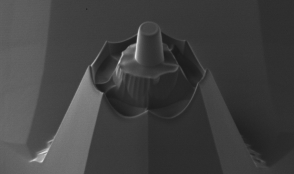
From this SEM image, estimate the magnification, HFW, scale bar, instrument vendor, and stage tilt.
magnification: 12 K X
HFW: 25.3 µm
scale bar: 5000 nm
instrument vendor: FEI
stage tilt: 45°